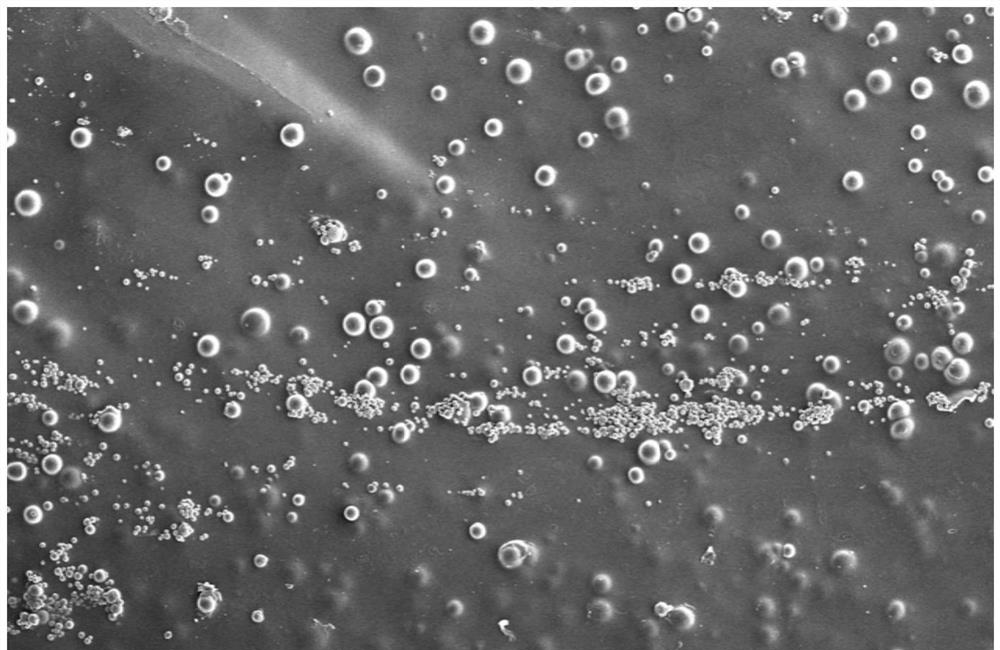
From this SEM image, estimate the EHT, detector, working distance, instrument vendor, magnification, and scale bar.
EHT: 15 kV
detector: ETD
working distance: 9.6 mm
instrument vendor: FEI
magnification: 0.1 K X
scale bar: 500000 nm